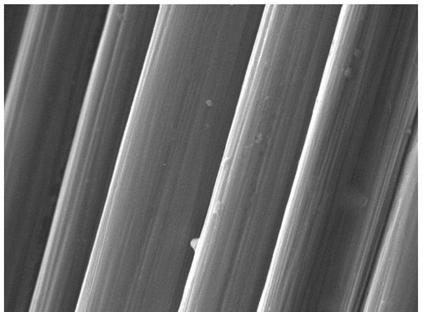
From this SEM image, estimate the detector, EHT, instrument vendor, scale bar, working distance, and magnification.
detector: SE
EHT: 10 kV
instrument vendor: Tescan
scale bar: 5000 nm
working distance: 15.45 mm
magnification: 10 K X